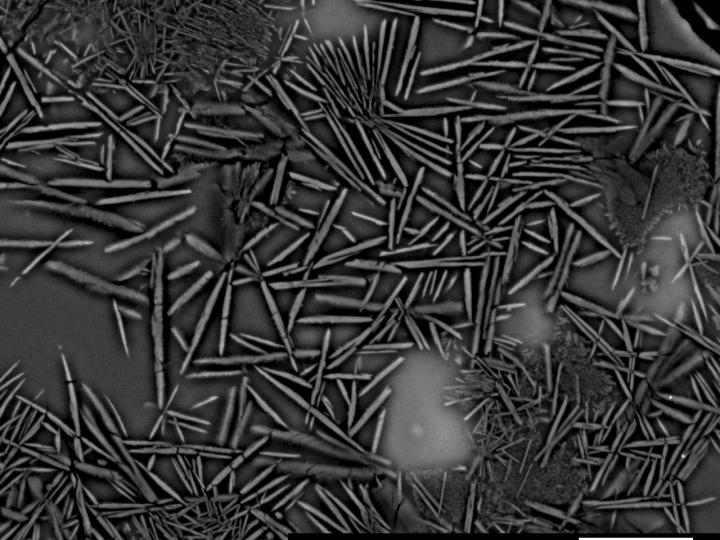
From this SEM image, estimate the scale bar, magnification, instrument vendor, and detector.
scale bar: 10000 nm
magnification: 1.9 K X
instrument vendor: JEOL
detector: COMP